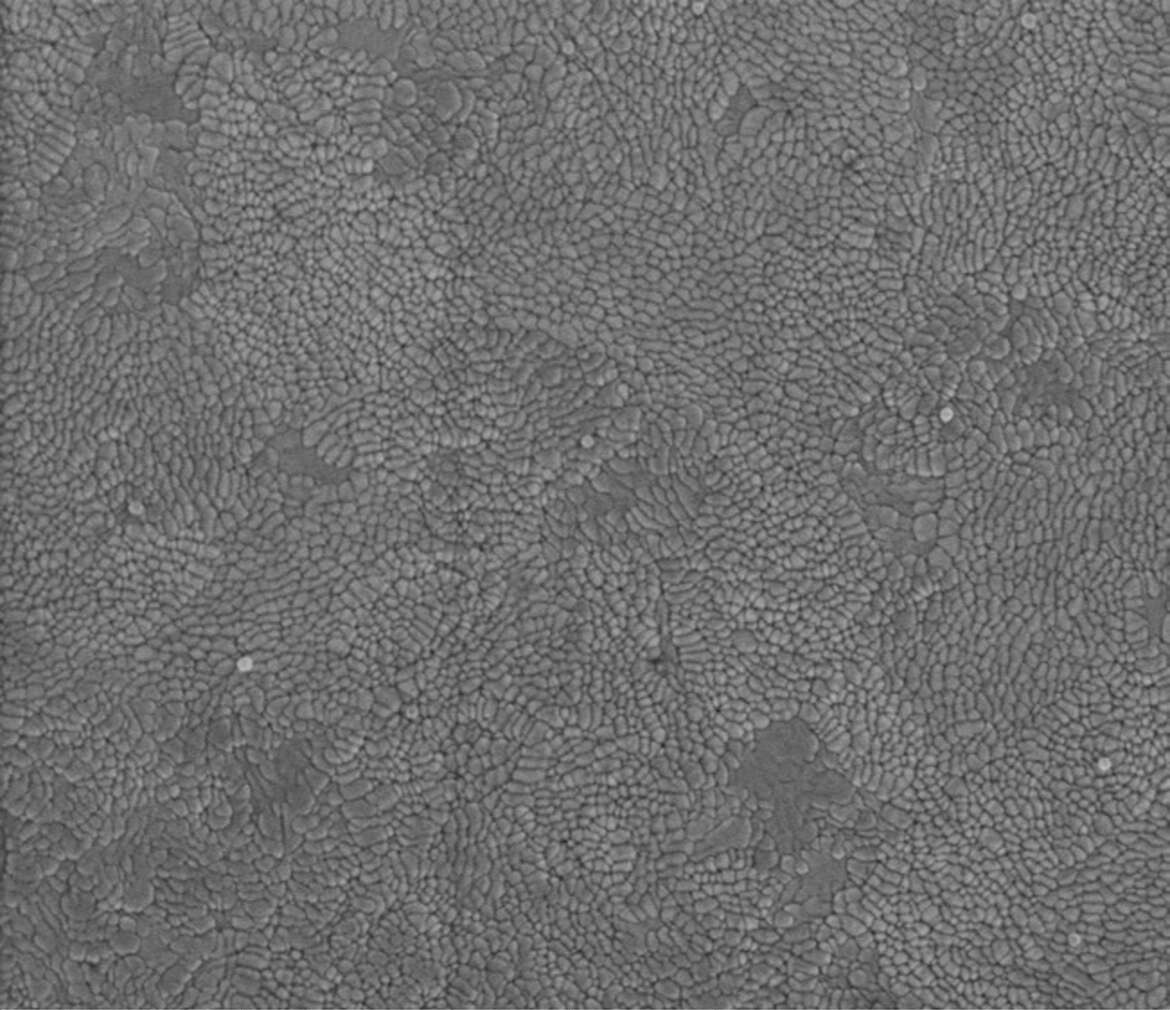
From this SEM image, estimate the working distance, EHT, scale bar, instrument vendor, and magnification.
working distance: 1.5 mm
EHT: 1 kV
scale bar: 500 nm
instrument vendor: FEI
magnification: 159.382 K X